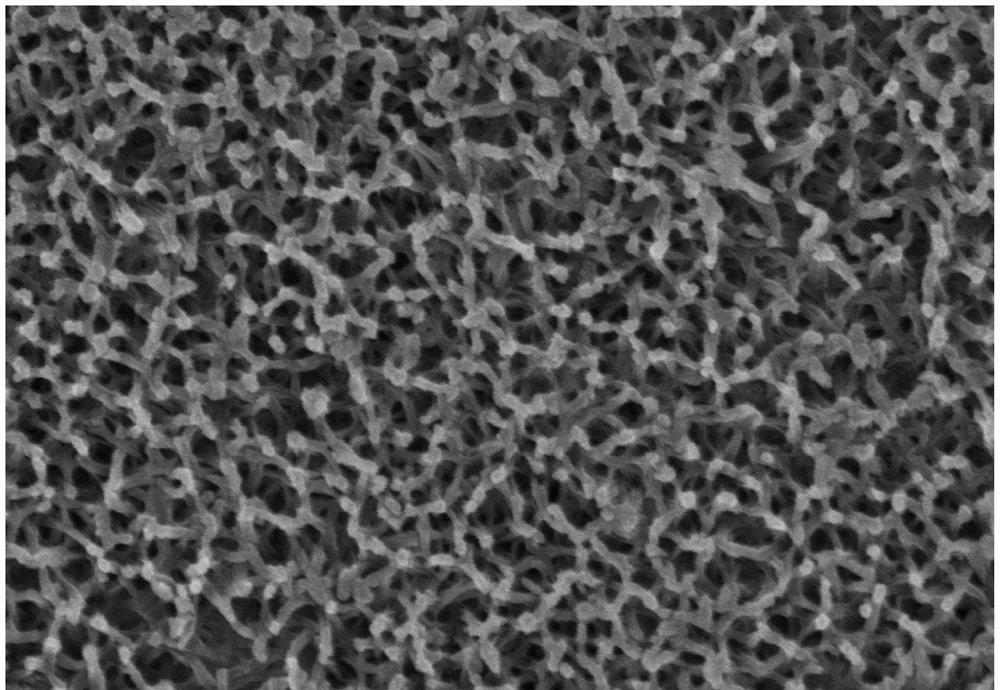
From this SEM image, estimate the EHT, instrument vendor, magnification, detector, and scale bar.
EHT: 8 kV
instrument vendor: Hitachi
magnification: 50 K X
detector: SE(M)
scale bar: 1000 nm